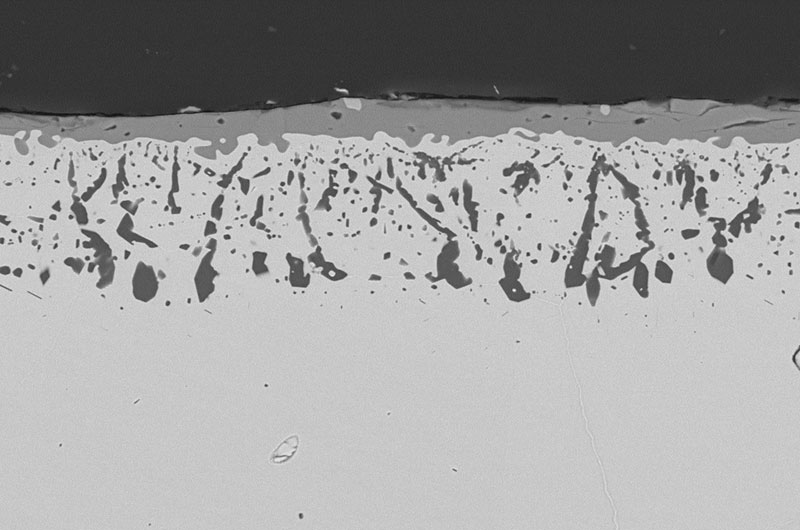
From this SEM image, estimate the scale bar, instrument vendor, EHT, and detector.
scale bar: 10000 nm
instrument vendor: other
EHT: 25 kV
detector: QBSD